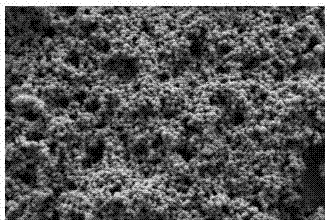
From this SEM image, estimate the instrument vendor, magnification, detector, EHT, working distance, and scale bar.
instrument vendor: Zeiss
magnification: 20 K X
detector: SE2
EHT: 1 kV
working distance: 4.1 mm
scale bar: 1000 nm